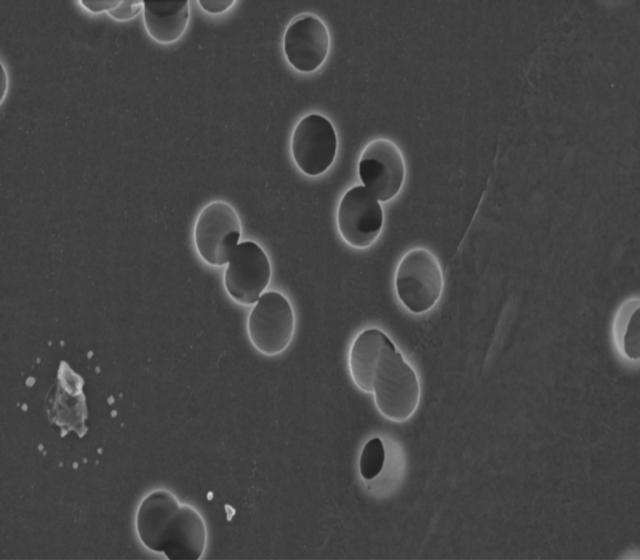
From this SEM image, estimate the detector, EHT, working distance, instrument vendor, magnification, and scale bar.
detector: InLens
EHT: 3 kV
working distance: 3 mm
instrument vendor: Zeiss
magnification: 30 K X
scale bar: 1000 nm